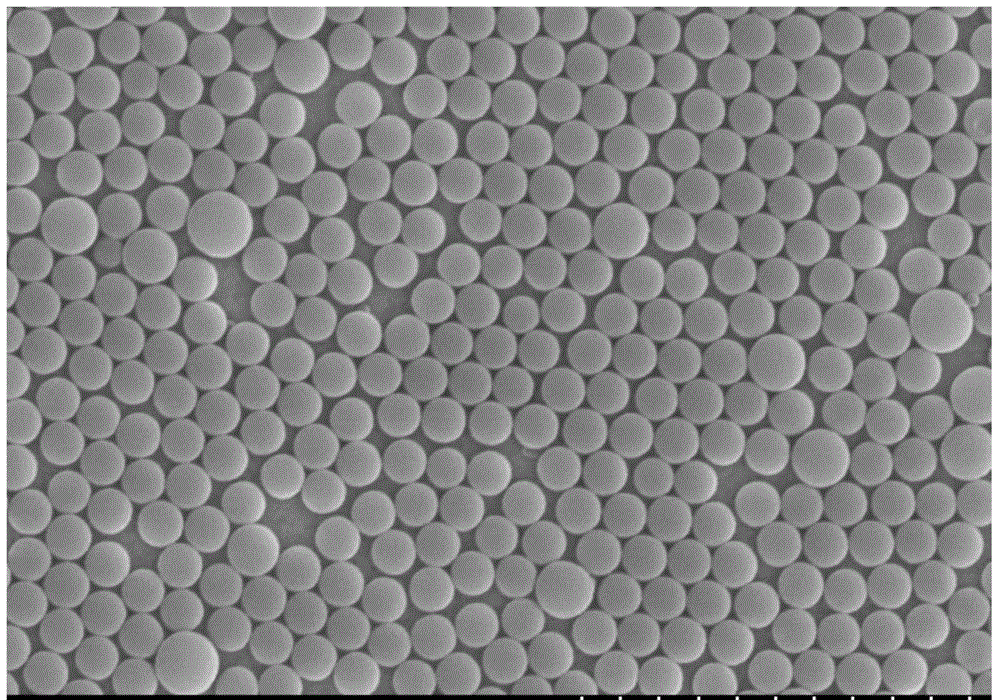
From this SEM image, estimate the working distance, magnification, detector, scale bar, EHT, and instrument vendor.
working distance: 13.3 mm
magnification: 10 K X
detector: SE(M,0)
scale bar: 5000 nm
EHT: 15 kV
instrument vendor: Hitachi